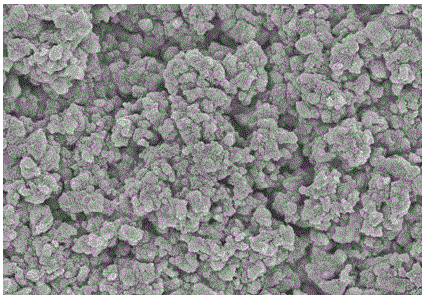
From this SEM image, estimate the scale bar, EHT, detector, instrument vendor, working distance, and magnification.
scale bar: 5000 nm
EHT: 5 kV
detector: SE(M)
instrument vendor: Hitachi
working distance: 8.9 mm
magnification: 10 K X